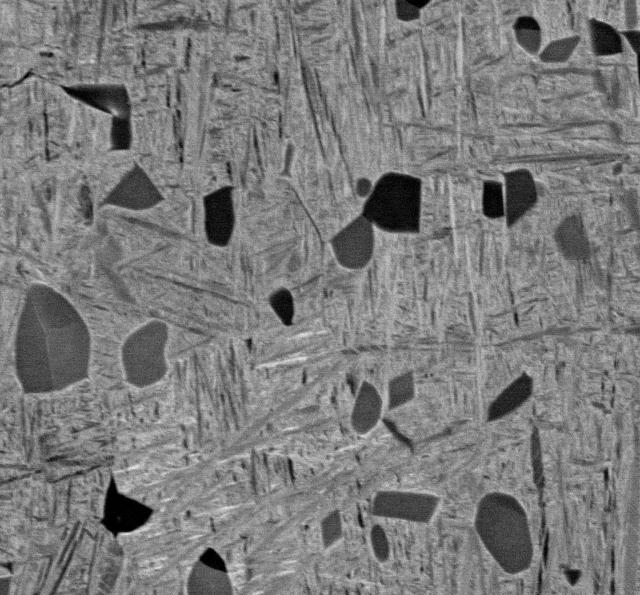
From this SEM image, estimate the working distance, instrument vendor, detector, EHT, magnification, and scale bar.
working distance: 10 mm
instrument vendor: JEOL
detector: COMPO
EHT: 15 kV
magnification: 2 K X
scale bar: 10000 nm